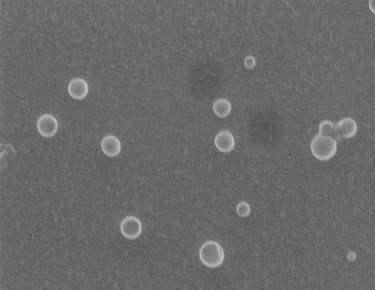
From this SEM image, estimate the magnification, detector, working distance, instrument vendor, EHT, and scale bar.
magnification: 50 K X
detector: SEI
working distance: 10.1 mm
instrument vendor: JEOL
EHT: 15 kV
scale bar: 100 nm